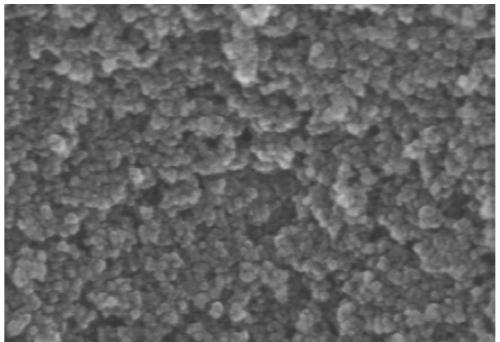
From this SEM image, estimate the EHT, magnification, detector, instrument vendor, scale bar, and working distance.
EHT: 15 kV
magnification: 200 K X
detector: SE(UL)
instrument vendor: Hitachi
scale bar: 200 nm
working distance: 9.2 mm